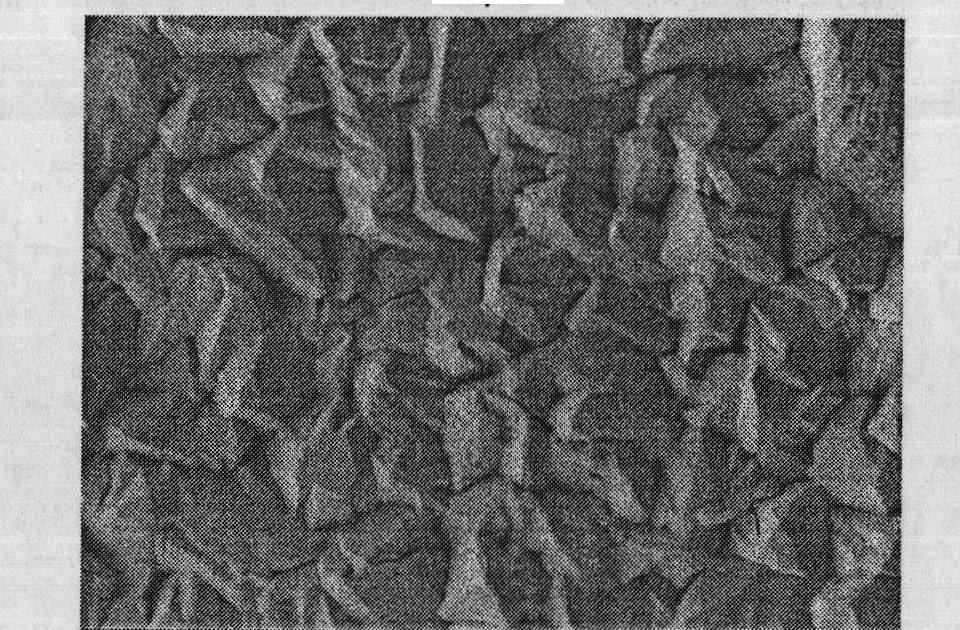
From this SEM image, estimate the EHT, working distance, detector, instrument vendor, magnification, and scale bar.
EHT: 3 kV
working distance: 7.5 mm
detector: LEI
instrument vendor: JEOL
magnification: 3 K X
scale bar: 1000 nm